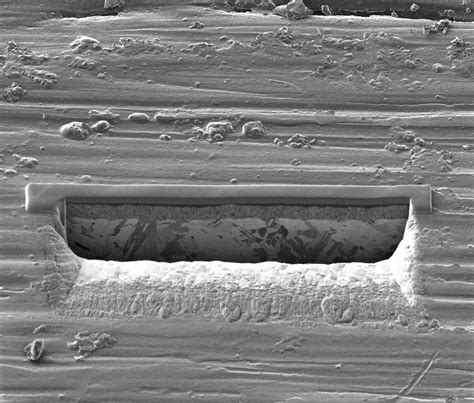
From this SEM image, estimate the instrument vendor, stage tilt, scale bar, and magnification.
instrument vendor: other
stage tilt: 45°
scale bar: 20000 nm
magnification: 6.5 K X